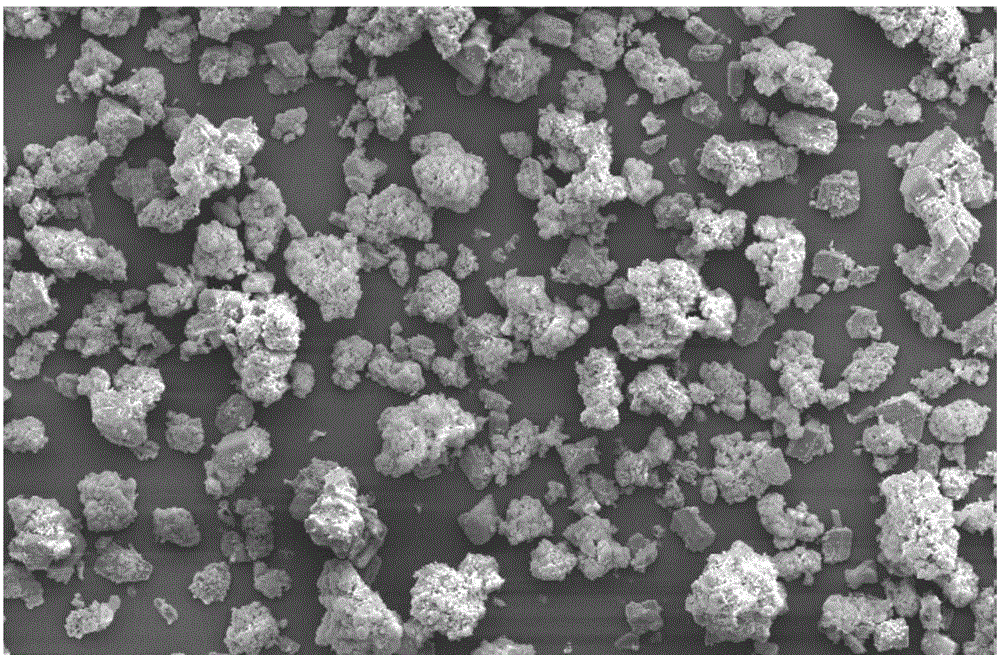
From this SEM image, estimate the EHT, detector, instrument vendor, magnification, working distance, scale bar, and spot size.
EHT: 10 kV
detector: SE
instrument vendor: FEI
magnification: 1 K X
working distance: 9.6 mm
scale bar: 50000 nm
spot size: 4